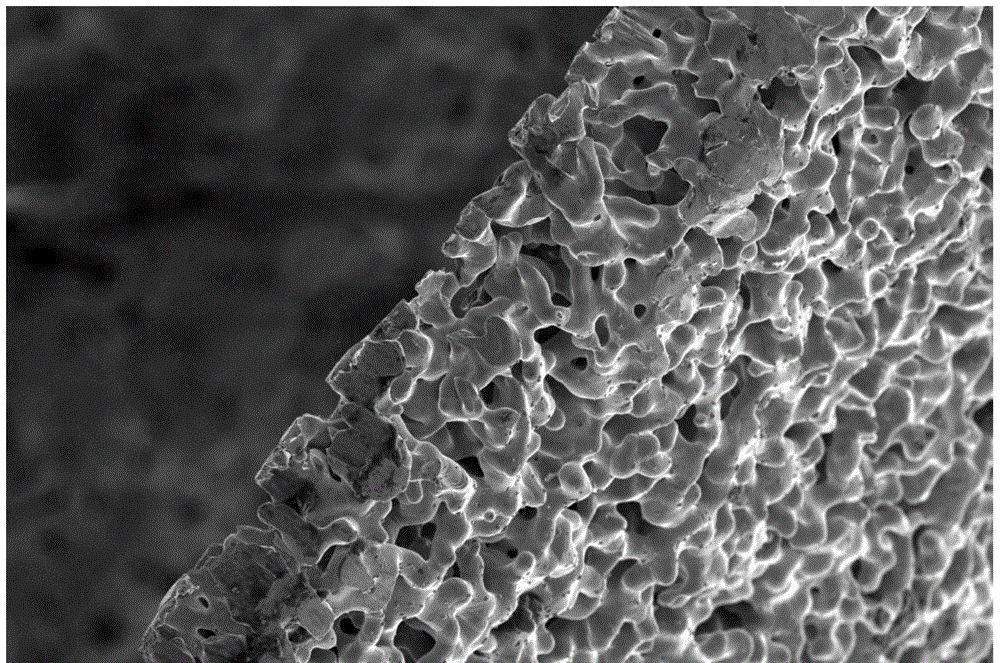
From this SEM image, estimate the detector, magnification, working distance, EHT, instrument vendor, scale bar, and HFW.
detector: SE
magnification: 1.5 K X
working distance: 9.5 mm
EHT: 5 kV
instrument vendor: FEI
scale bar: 50000 nm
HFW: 138 µm